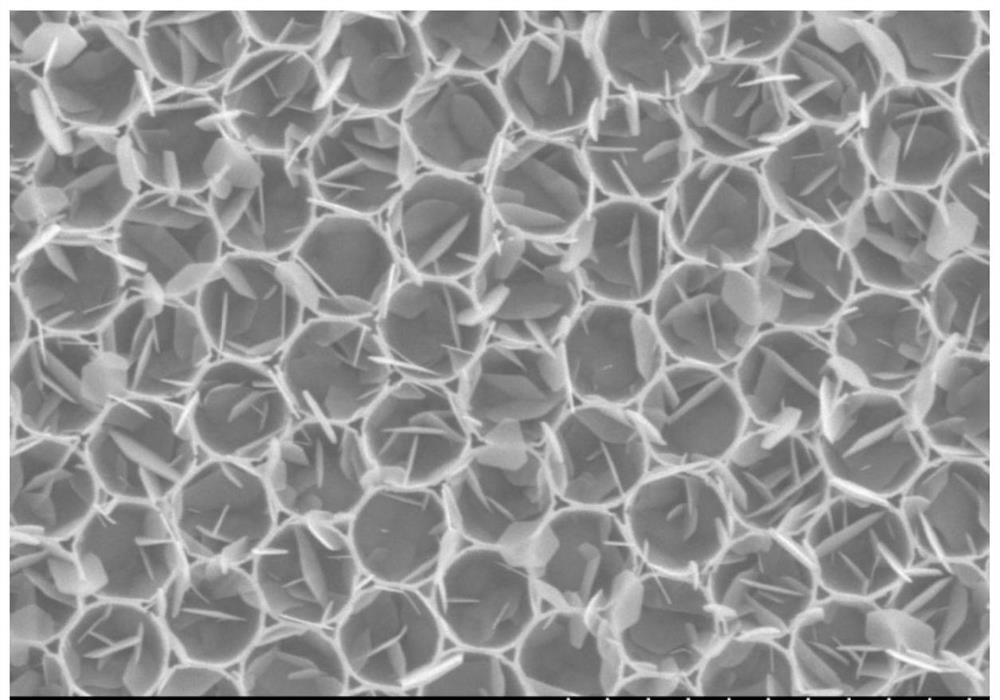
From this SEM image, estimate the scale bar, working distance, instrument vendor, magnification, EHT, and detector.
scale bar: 4000 nm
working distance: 31.6 mm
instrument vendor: Hitachi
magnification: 13 K X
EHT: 10 kV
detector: SE